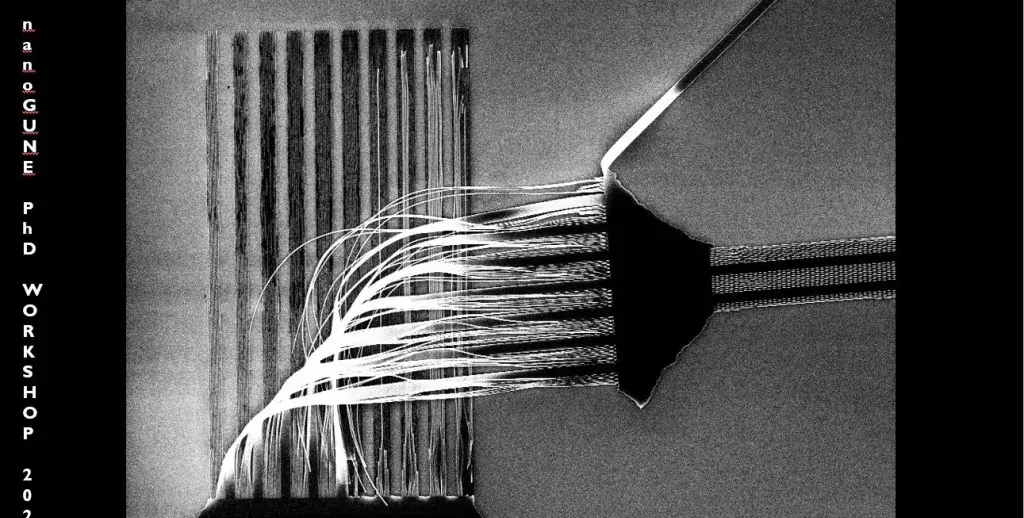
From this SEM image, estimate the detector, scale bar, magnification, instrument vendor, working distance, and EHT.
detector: InLens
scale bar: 10000 nm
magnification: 1.38 K X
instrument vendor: Zeiss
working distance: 10.2 mm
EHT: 10 kV